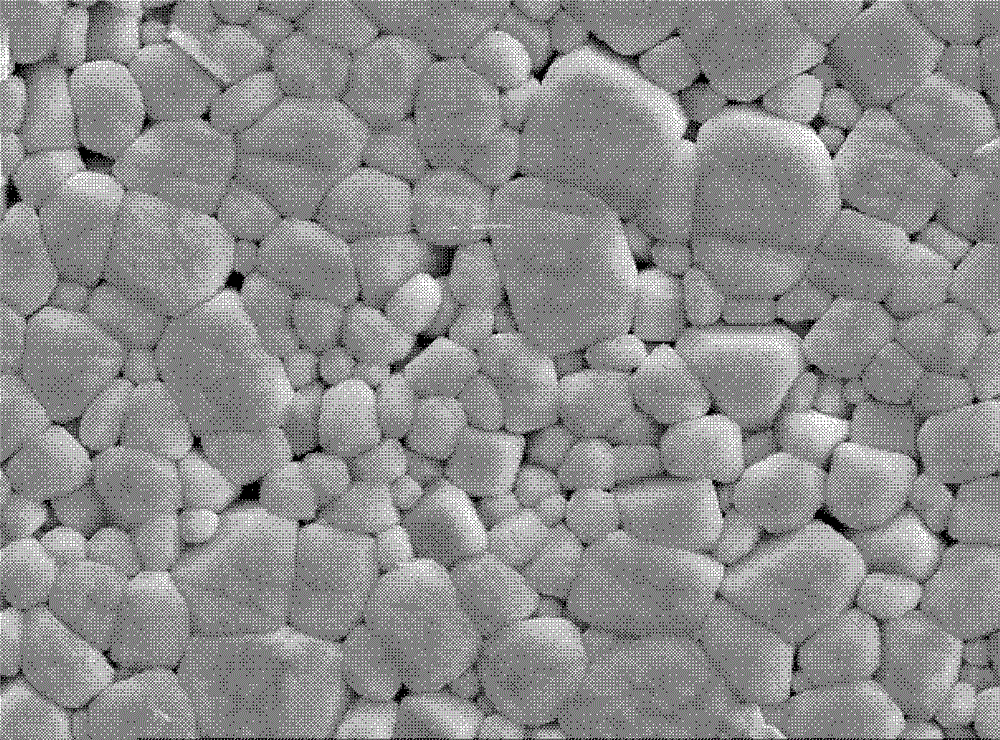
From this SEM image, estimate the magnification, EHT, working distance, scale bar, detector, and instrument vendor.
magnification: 3 K X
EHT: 20 kV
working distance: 11 mm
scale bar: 1000 nm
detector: SEI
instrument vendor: JEOL